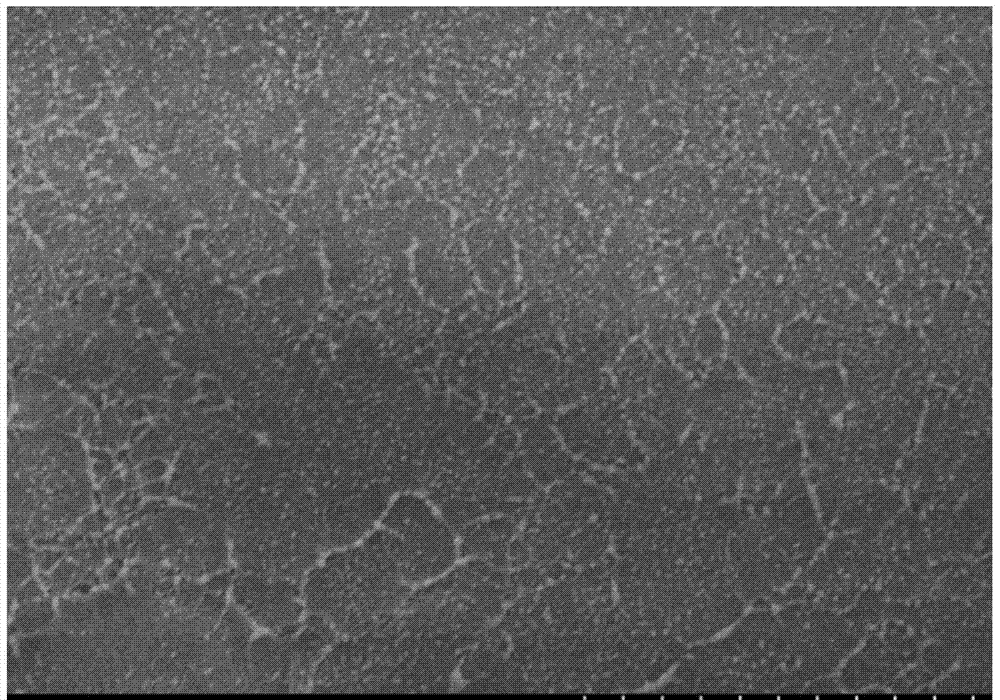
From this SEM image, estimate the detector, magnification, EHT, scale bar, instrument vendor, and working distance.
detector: SE(M)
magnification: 10 K X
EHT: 5 kV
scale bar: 5000 nm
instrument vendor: Hitachi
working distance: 9 mm